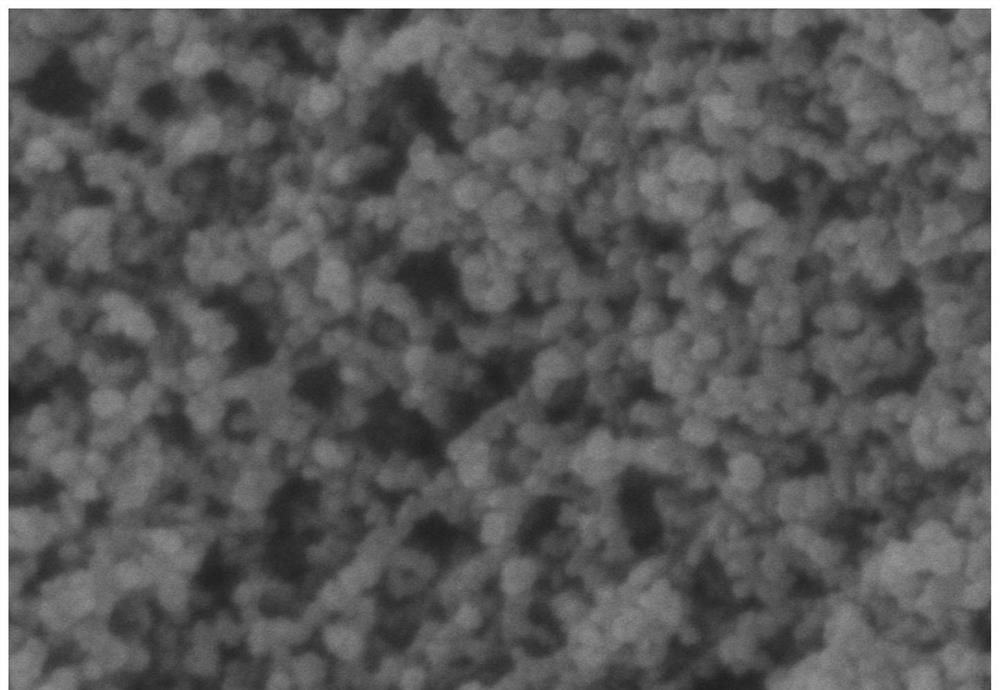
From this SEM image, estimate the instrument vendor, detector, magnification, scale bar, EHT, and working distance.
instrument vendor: Hitachi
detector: SE(U)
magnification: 150 K X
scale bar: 300 nm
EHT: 5 kV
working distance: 9.7 mm